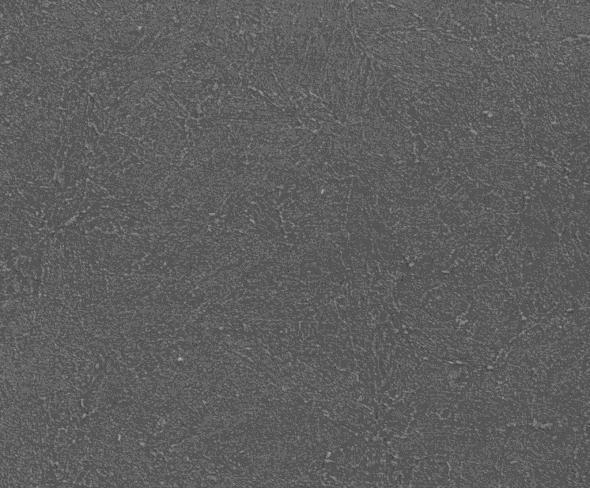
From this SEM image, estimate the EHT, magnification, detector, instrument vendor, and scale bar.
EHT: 20 kV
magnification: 5 K X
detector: SE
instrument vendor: Tescan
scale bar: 10000 nm